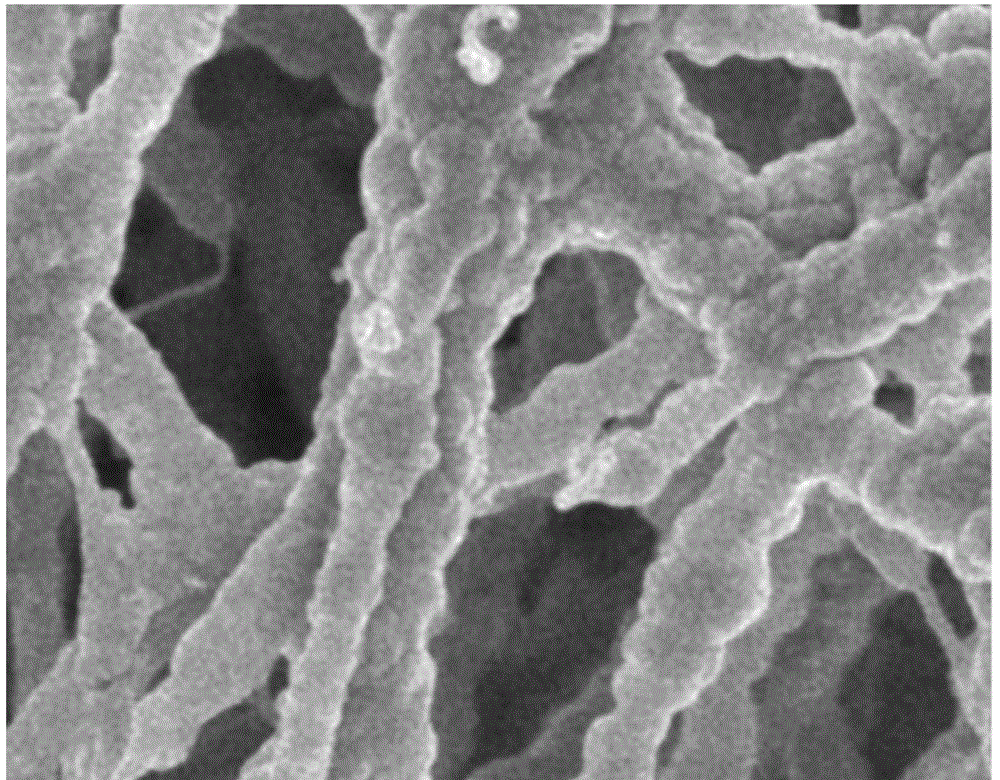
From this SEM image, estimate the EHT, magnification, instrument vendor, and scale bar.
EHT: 5 kV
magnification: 10 K X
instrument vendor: JEOL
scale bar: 1000 nm